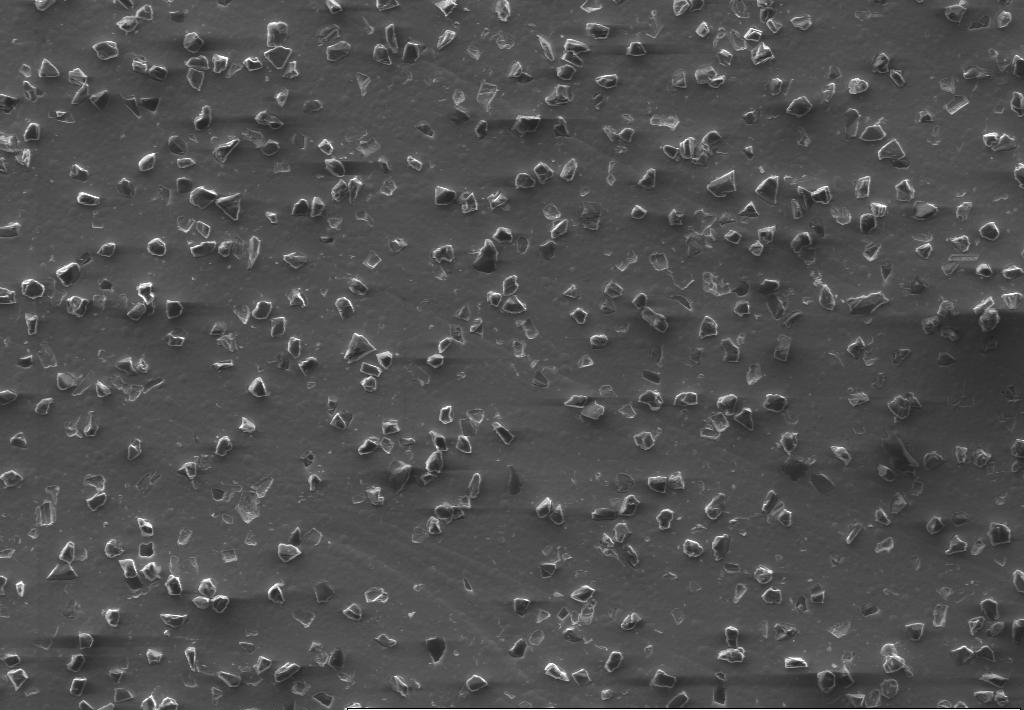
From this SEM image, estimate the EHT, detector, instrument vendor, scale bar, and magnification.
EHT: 15 kV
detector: SE1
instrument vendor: Zeiss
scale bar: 20000 nm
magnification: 0.5 K X